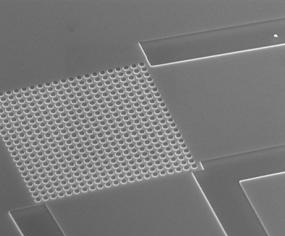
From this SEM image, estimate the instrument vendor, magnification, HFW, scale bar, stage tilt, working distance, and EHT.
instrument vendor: FEI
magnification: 12 K X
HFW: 21.3 µm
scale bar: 5000 nm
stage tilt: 52°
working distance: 6.1 mm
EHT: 18 kV